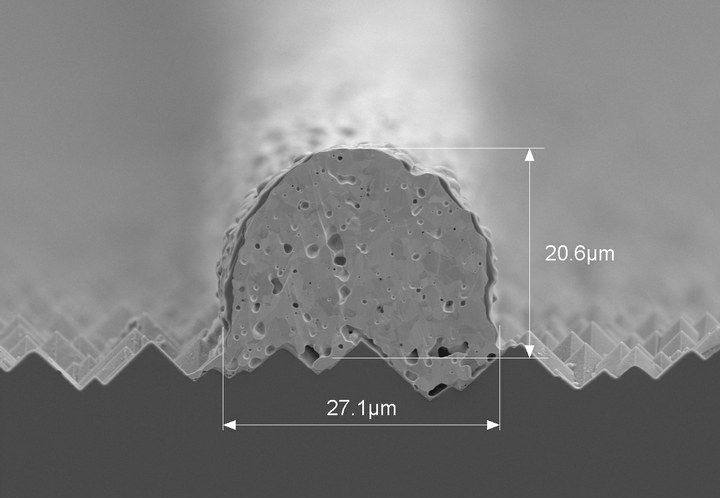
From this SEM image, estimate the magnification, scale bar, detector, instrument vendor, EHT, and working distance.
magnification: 1.8 K X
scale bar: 30000 nm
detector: SE(M)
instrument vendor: Hitachi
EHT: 5 kV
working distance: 14.9 mm